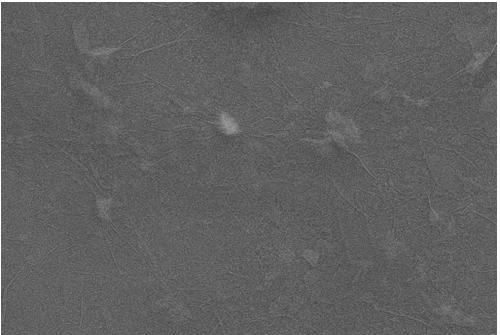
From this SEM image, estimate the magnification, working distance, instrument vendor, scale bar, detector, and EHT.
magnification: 5 K X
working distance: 8.1 mm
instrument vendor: Zeiss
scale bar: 2000 nm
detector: SE2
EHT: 15 kV